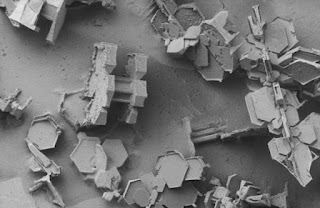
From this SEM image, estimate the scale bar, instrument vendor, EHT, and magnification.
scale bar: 1000000 nm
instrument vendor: JEOL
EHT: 2 kV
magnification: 0.03 K X